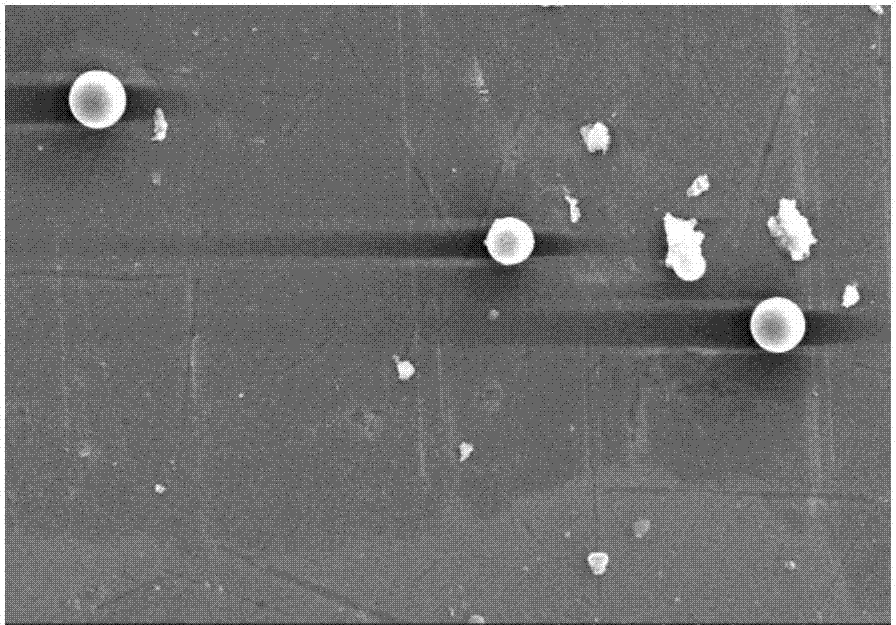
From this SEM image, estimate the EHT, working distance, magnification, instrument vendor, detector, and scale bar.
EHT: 15 kV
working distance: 25.4 mm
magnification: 1 K X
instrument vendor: Hitachi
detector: SE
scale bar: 50000 nm